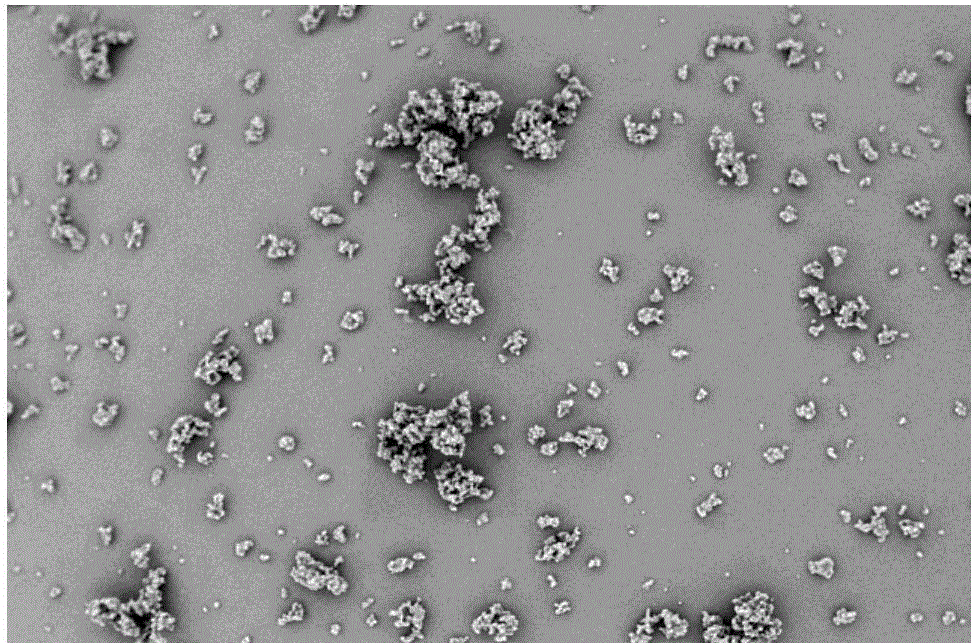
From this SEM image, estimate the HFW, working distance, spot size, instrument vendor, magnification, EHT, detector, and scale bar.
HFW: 8.29 µm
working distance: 6.1 mm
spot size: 2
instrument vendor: FEI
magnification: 50 K X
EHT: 1 kV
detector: CBS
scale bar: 3000 nm